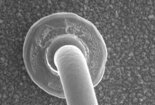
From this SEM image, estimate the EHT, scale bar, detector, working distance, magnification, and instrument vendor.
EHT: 25 kV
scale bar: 40000 nm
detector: SE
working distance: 11 mm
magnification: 1.3 K X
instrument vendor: Hitachi